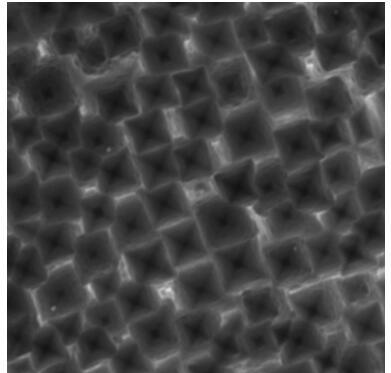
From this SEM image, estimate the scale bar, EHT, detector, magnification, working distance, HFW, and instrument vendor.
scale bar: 2000 nm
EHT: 20 kV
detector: SE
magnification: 20 K X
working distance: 15.18 mm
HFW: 10.4 µm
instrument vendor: Tescan